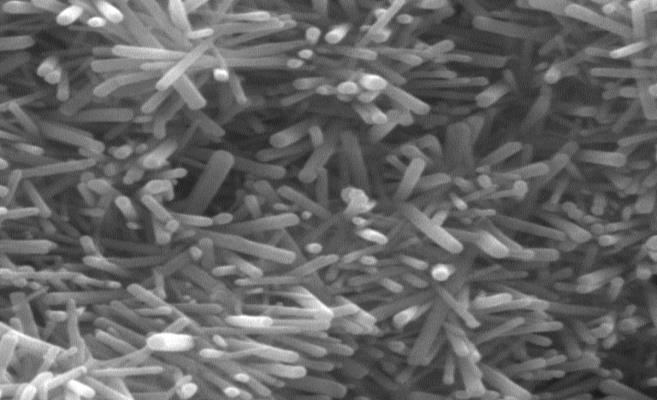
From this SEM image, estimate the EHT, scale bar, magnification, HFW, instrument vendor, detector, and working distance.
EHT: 20 kV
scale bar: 500 nm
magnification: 65 K X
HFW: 3.19 µm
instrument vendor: Tescan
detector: SE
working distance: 7.52 mm